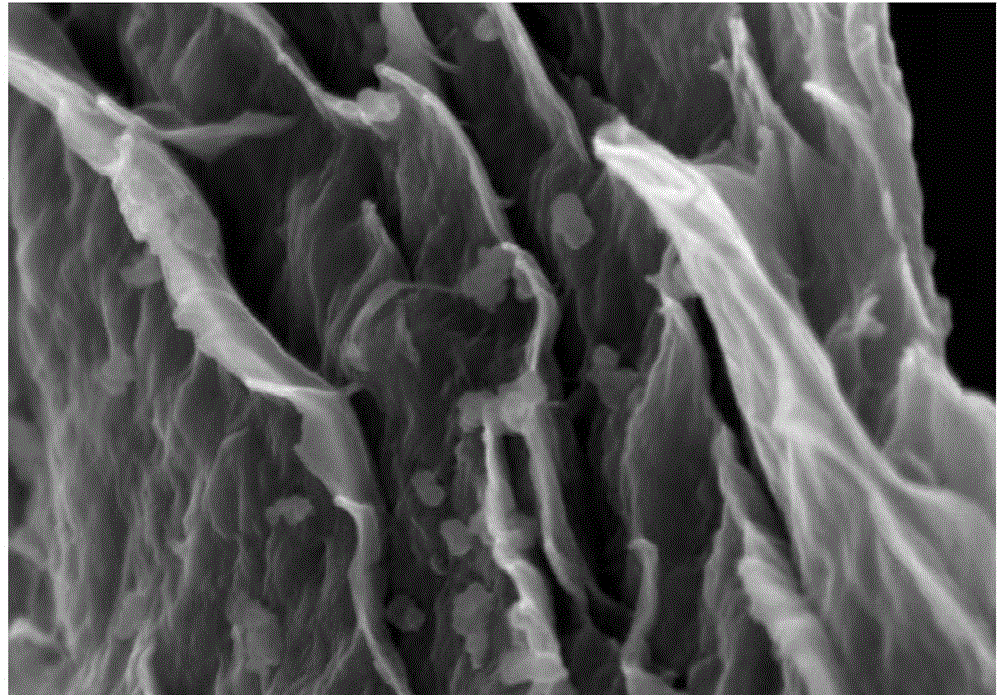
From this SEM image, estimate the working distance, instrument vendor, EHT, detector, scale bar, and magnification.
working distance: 4.7 mm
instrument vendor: Zeiss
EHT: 5 kV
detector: InLens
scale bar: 200 nm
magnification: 50 K X